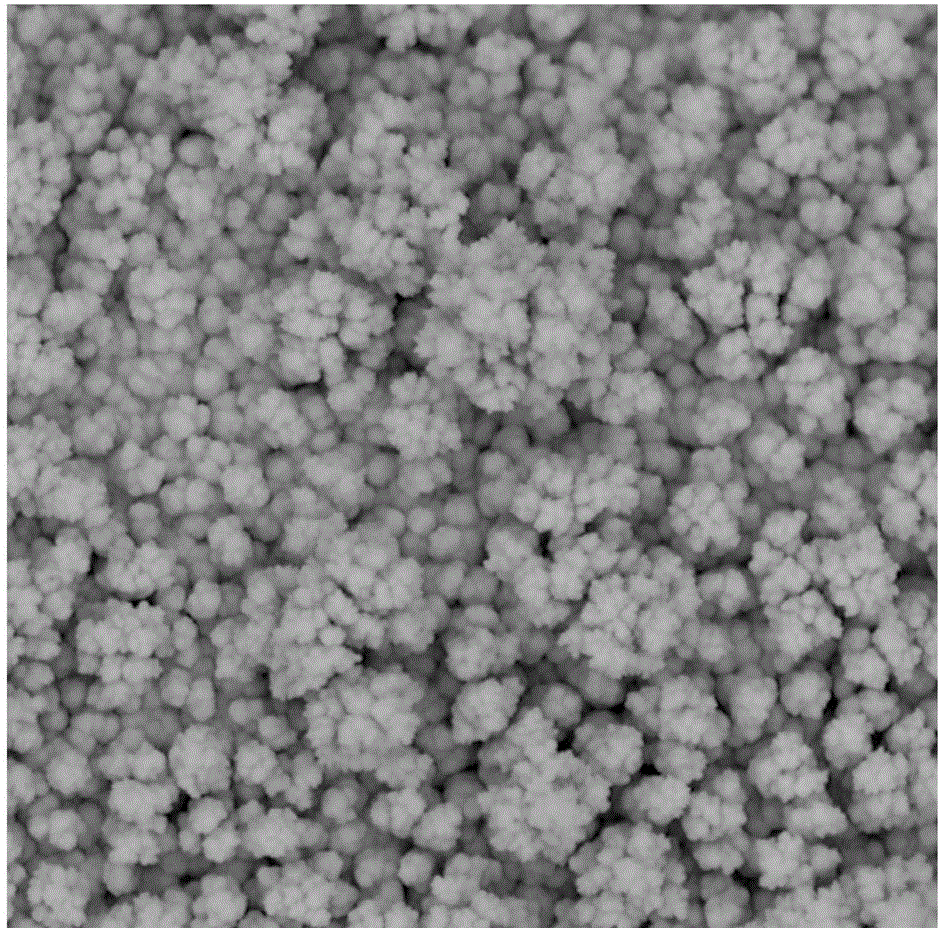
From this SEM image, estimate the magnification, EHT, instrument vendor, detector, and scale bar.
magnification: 10 K X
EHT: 15 kV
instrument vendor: other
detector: BSD Full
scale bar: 8000 nm